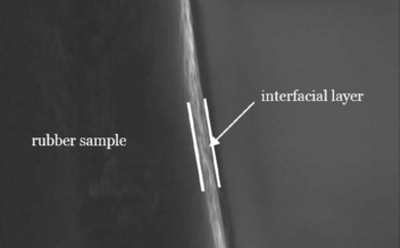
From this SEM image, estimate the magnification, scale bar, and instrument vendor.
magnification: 8 K X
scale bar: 2000 nm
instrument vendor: JEOL